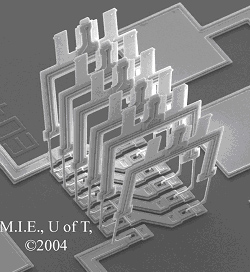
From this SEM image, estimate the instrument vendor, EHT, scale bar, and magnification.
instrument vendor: JEOL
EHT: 20 kV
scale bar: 100000 nm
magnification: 0.3 K X